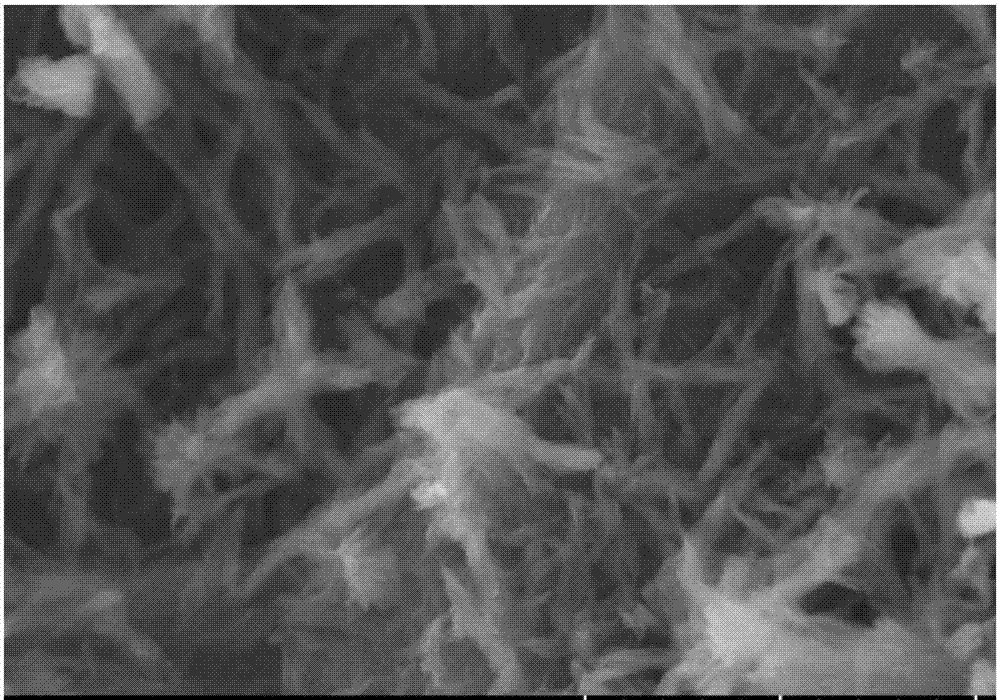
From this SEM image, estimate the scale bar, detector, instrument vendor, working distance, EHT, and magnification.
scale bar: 5000 nm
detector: SE(UL)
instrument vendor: Hitachi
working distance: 9.5 mm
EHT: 10 kV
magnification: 10 K X